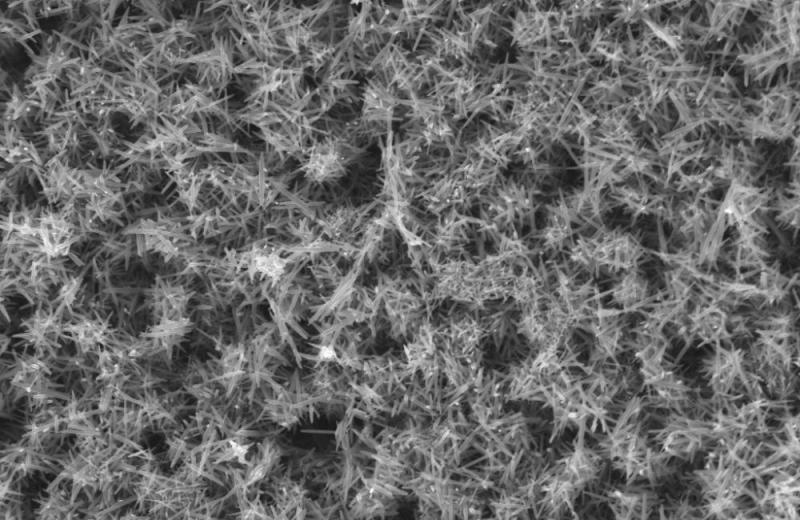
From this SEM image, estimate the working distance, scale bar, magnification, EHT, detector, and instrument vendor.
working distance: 6 mm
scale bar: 2000 nm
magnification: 10 K X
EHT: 10 kV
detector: InLens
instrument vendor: Zeiss